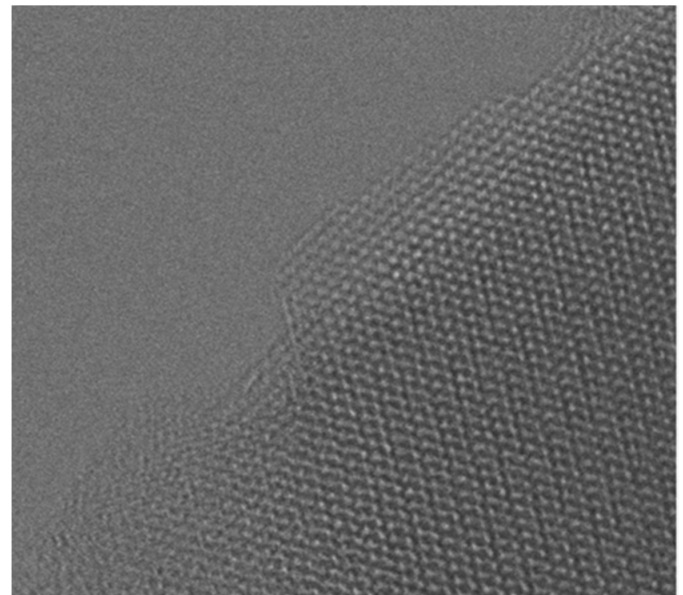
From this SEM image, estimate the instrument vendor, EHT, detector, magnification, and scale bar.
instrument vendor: JEOL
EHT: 200 kV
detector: BF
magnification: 1400 K X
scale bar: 5 nm